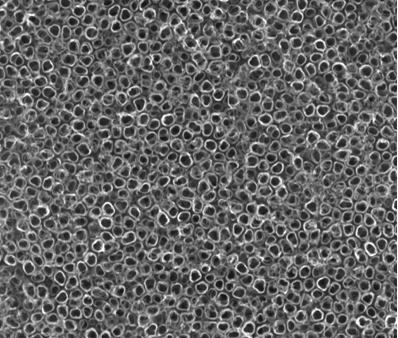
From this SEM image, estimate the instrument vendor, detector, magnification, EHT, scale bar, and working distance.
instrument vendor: FEI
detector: TLD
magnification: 100 K X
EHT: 20 kV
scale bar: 500 nm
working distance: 6 mm